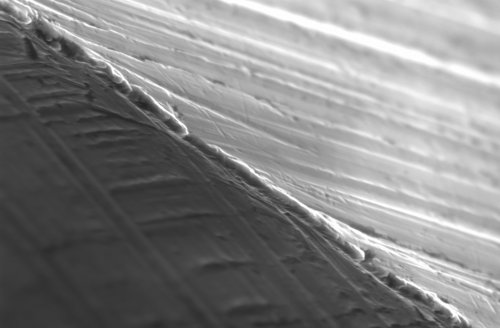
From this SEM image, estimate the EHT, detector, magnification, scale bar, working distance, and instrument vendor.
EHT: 20 kV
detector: SE1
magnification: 2 K X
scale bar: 2000 nm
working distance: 10.5 mm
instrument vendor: Zeiss